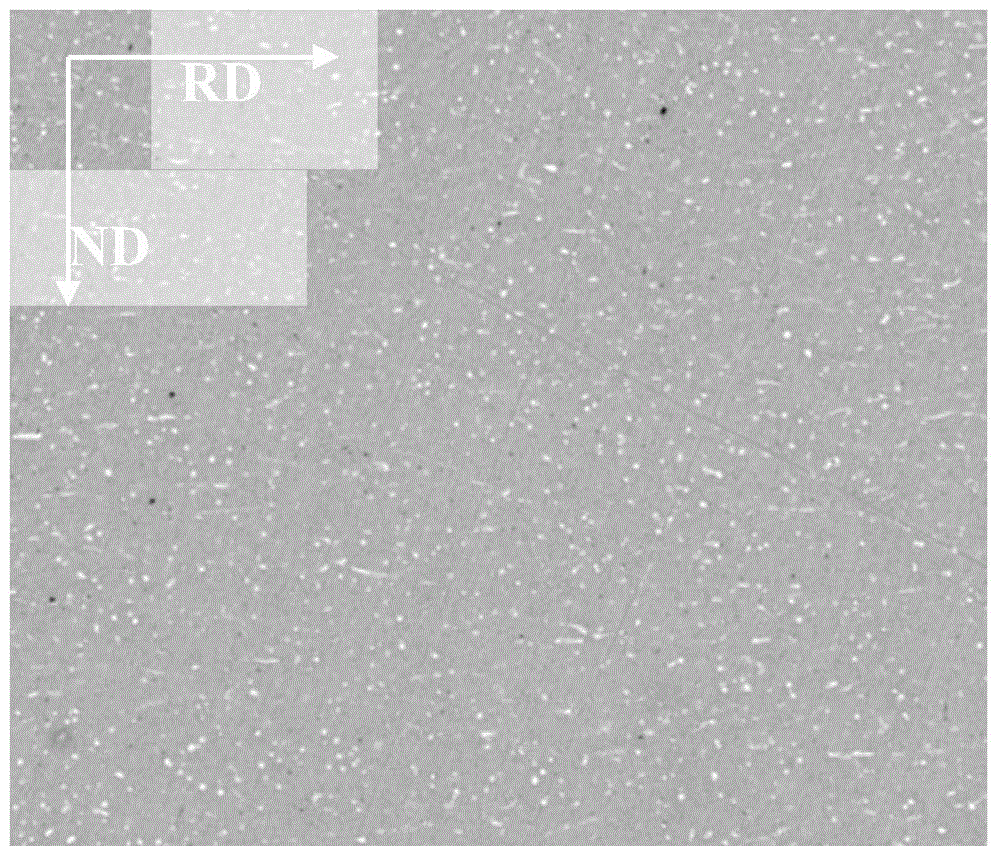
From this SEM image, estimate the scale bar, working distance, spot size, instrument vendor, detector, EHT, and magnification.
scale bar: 50000 nm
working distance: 10 mm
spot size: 6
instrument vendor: FEI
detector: DualBSD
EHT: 20 kV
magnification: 2 K X